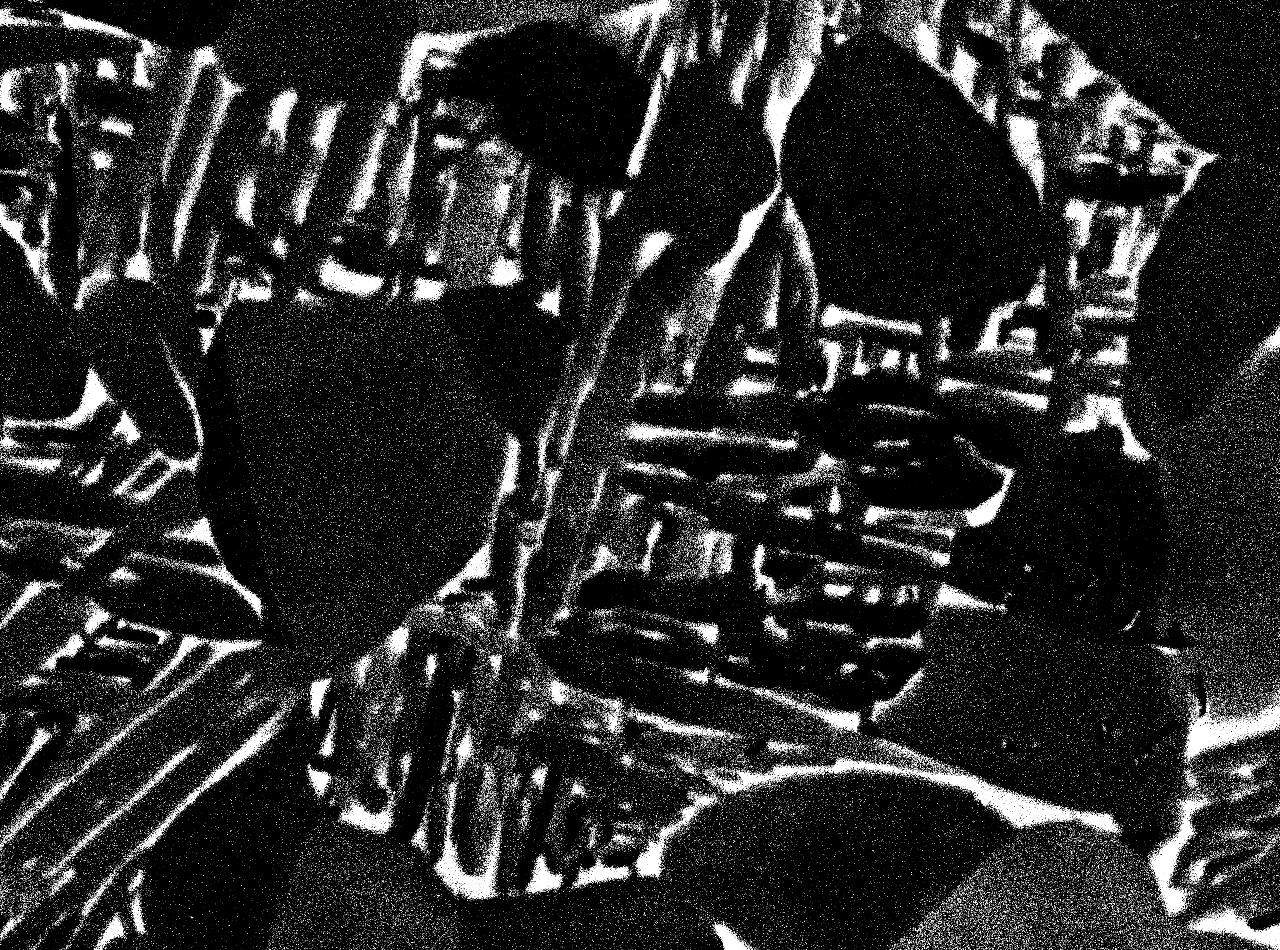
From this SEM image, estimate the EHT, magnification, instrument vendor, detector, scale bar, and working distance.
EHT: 15 kV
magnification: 5 K X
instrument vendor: JEOL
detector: COMPO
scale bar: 1000 nm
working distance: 10 mm